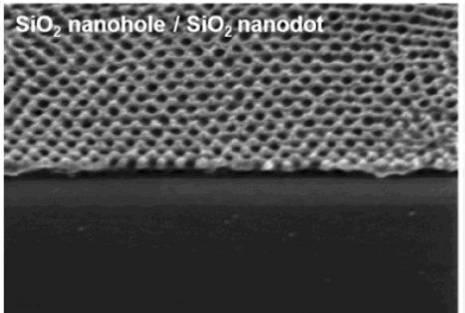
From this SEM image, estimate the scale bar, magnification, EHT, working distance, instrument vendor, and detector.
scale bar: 500 nm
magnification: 100 K X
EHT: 1 kV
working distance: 1.7 mm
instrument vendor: Hitachi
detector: SE(U)